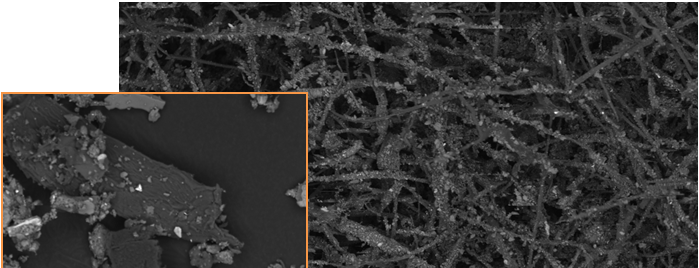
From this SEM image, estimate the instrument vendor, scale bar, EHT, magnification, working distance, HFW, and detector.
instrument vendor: FEI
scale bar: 300000 nm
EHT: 10 kV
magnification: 0.131 K X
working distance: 17.7 mm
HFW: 1580 µm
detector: BSED